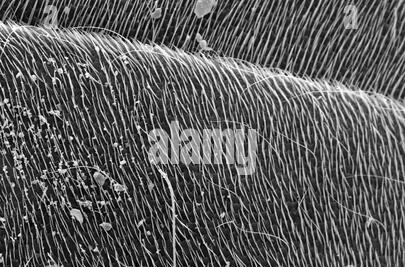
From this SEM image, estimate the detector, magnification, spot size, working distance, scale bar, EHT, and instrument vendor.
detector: SE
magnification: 0.185 K X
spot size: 3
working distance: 7.3 mm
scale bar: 200000 nm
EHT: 30 kV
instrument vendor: FEI